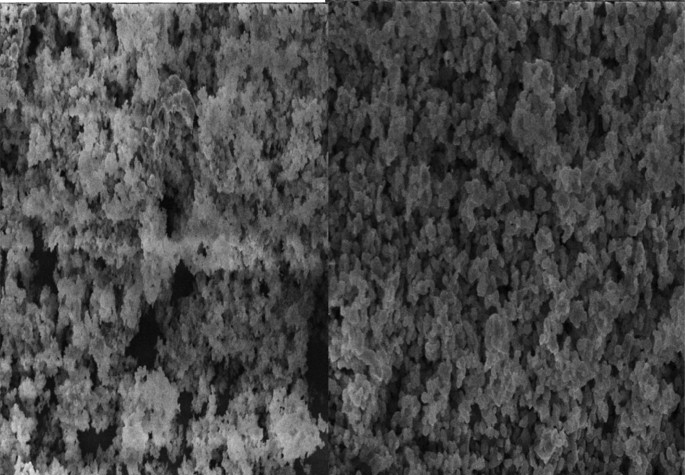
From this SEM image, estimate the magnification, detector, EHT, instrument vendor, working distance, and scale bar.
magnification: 1 K X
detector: SE1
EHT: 15 kV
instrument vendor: Zeiss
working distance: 31 mm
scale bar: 10000 nm